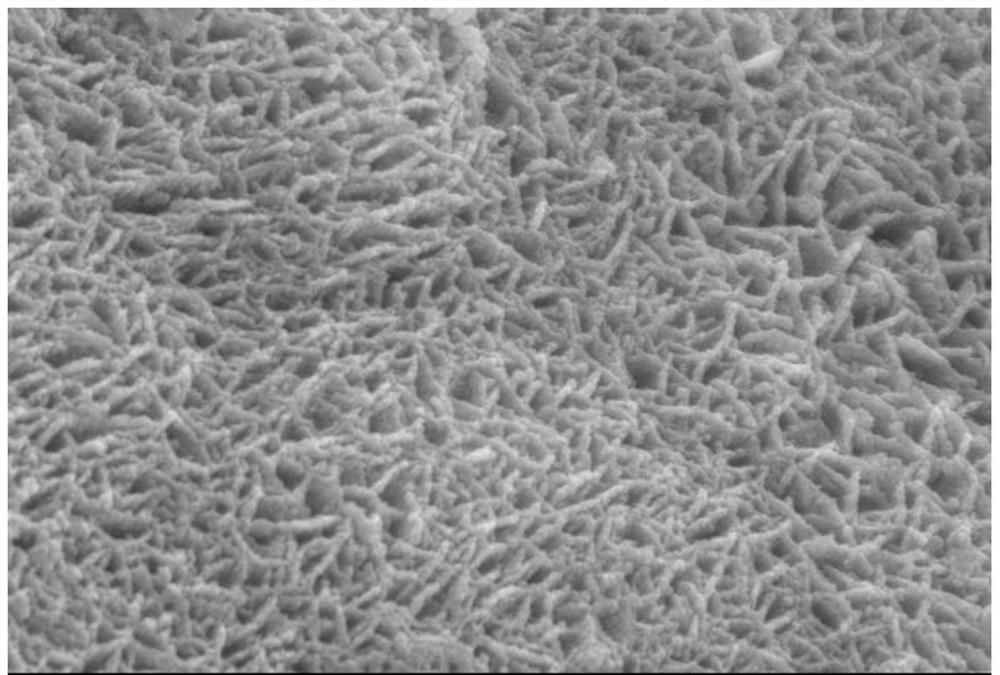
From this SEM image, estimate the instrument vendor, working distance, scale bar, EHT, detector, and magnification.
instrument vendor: Hitachi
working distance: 15.6 mm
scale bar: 2000 nm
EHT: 20 kV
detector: SE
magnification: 15 K X